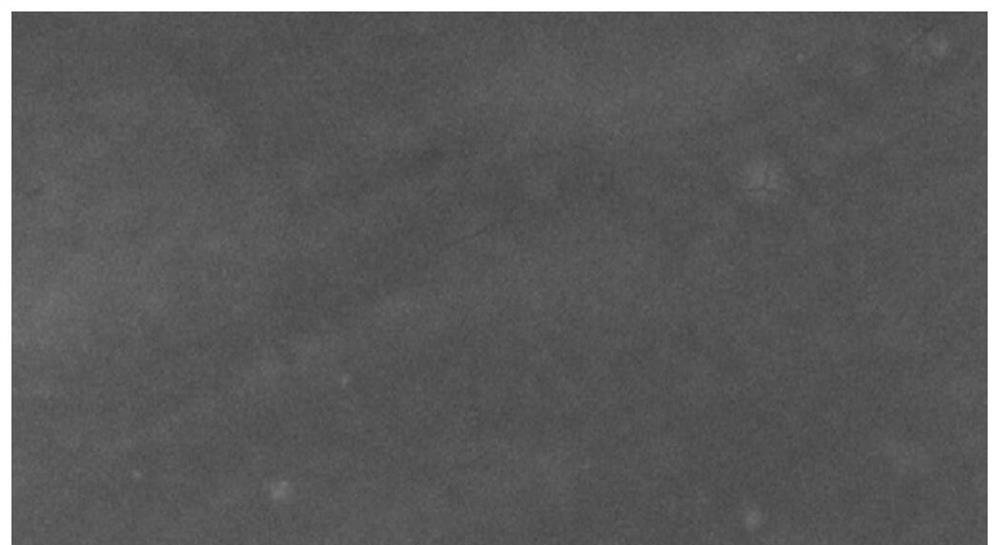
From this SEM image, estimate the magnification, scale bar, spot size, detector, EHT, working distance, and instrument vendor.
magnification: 1.2 K X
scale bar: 50000 nm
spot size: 4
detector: ETD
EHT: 20 kV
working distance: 13.6 mm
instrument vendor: FEI